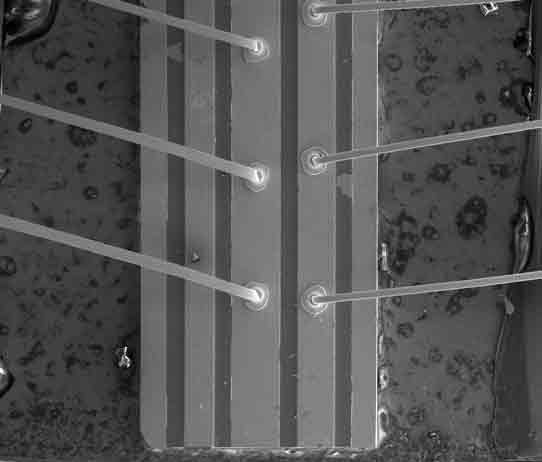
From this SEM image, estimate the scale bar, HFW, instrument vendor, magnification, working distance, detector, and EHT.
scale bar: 500000 nm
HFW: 1830 µm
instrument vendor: FEI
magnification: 0.07 K X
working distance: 8.3 mm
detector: ETD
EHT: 15 kV